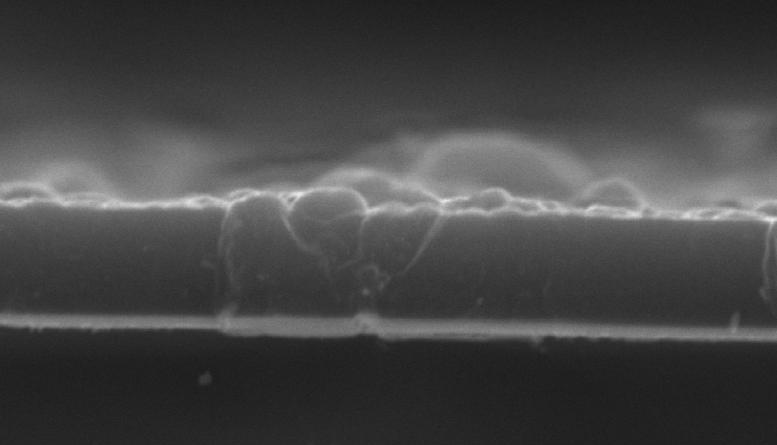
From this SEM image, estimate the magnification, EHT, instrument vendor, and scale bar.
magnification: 10 K X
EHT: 20 kV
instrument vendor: JEOL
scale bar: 1000 nm